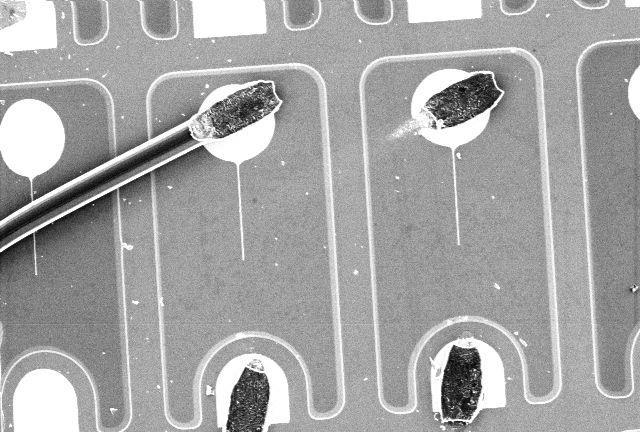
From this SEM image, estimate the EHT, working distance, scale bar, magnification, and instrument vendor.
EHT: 12.5 kV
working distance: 48 mm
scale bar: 100000 nm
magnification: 0.15 K X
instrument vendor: other